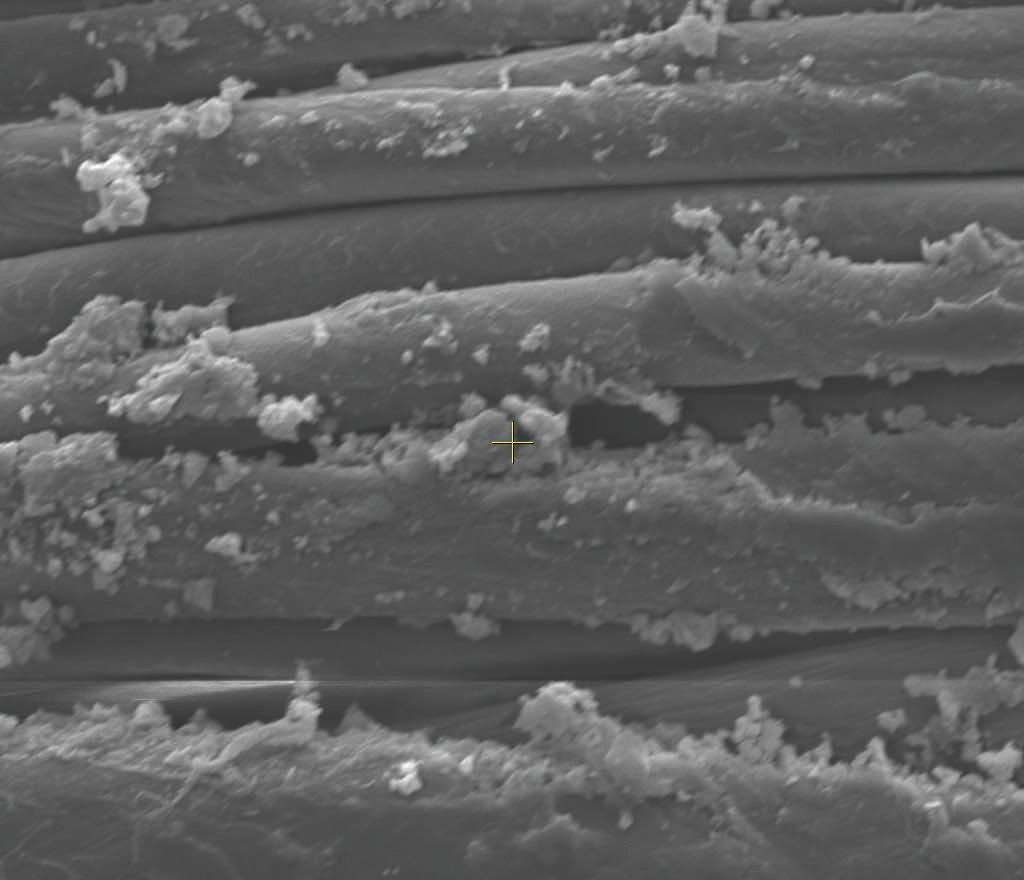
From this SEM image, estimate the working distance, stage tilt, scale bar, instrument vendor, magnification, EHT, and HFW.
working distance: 5.3 mm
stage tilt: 0°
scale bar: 10000 nm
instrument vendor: FEI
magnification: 5 K X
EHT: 10 kV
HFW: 59.7 µm